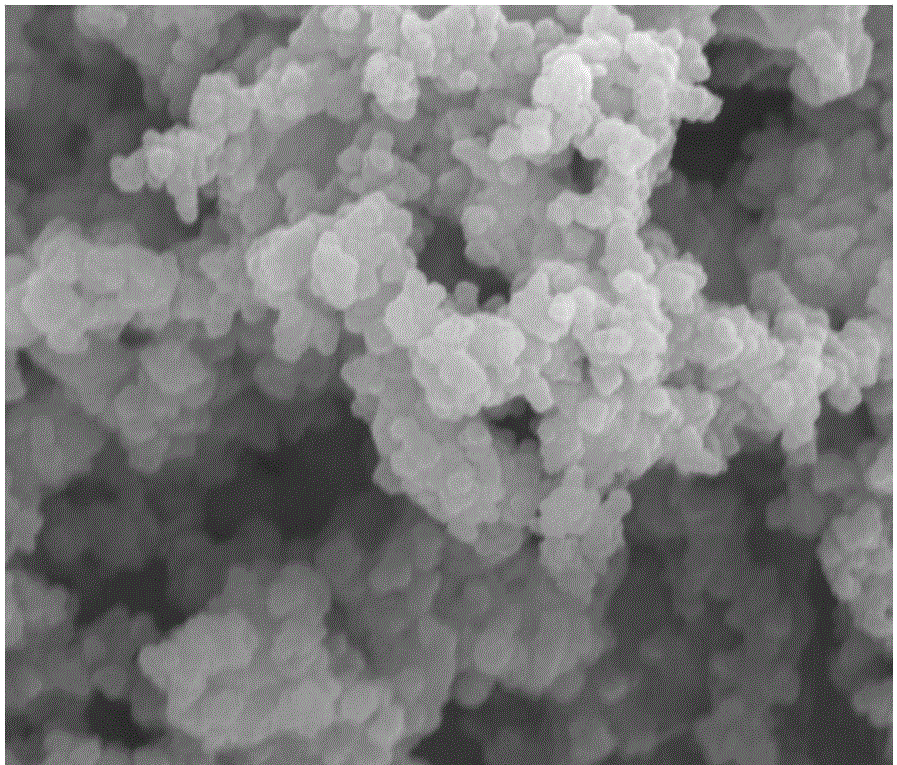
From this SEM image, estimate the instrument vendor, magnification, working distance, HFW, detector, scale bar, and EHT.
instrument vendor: FEI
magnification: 50 K X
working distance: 5 mm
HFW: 4.8 µm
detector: TLD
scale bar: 1000 nm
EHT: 10 kV